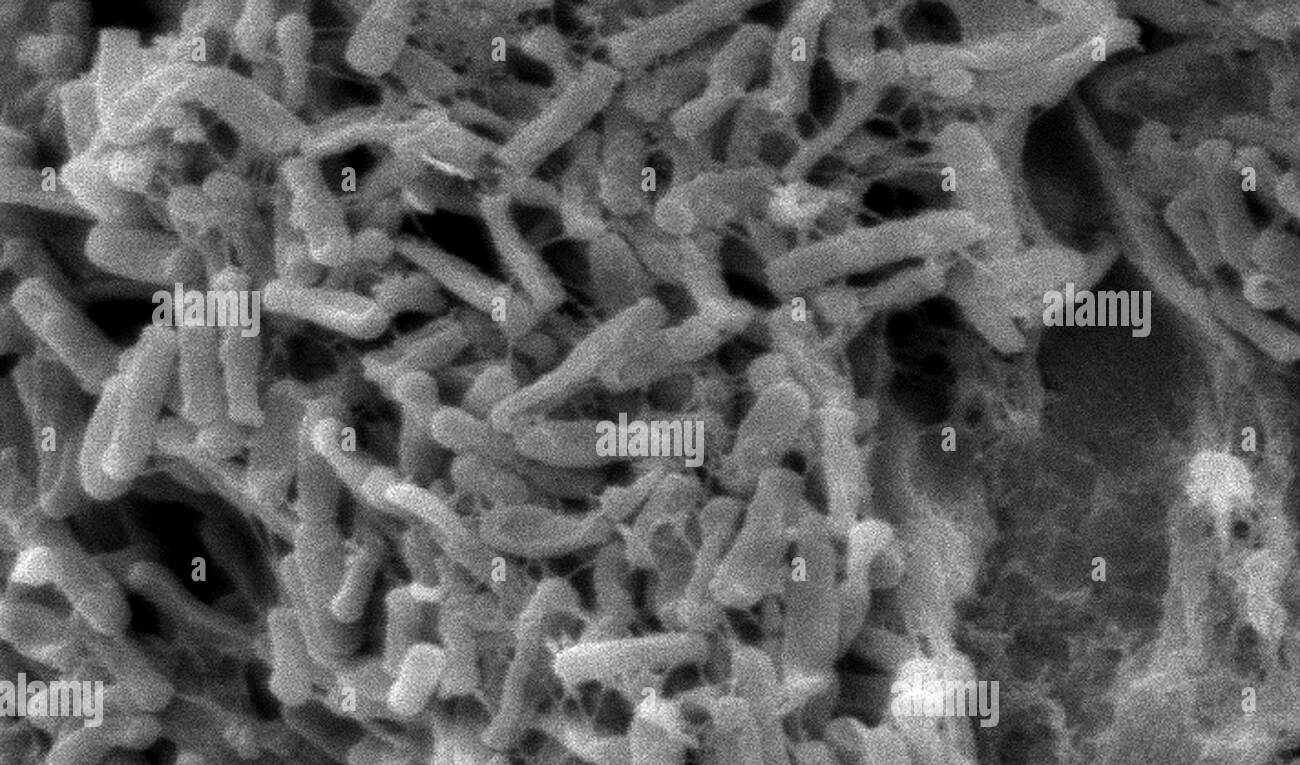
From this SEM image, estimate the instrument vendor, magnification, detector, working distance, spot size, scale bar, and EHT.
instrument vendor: FEI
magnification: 4.595 K X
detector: SE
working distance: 33.6 mm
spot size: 3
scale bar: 5000 nm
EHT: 10 kV